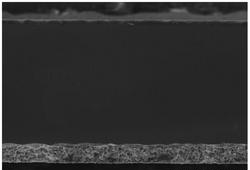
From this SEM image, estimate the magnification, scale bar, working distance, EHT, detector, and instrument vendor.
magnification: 0.35 K X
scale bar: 100000 nm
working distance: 6.5 mm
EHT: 20 kV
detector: SE(L)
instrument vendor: Hitachi